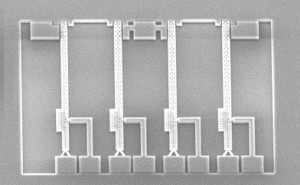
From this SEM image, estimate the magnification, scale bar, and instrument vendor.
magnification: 0.2 K X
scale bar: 150000 nm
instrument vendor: Hitachi